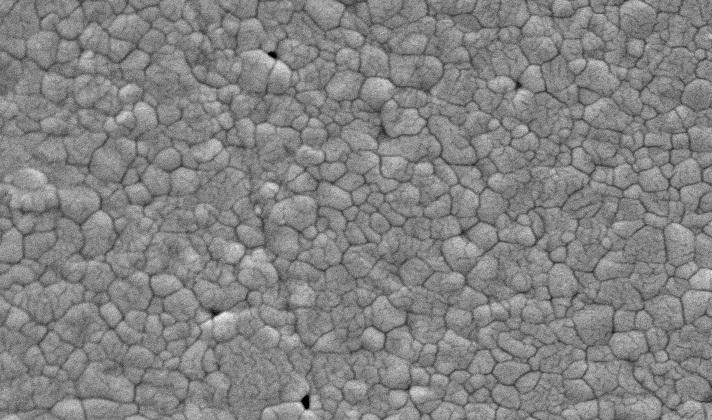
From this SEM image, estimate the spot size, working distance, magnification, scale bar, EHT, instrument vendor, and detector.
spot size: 3.4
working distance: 8.1 mm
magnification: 10 K X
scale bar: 2000 nm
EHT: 20 kV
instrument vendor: FEI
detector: SE